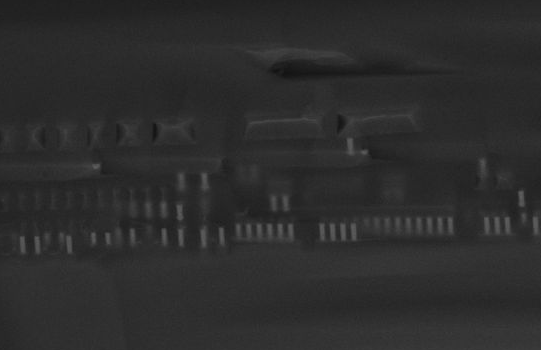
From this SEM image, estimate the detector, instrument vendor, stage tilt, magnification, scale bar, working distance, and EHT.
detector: InLens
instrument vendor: Zeiss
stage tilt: -15°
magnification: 11.47 K X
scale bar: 2000 nm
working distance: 4 mm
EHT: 15 kV